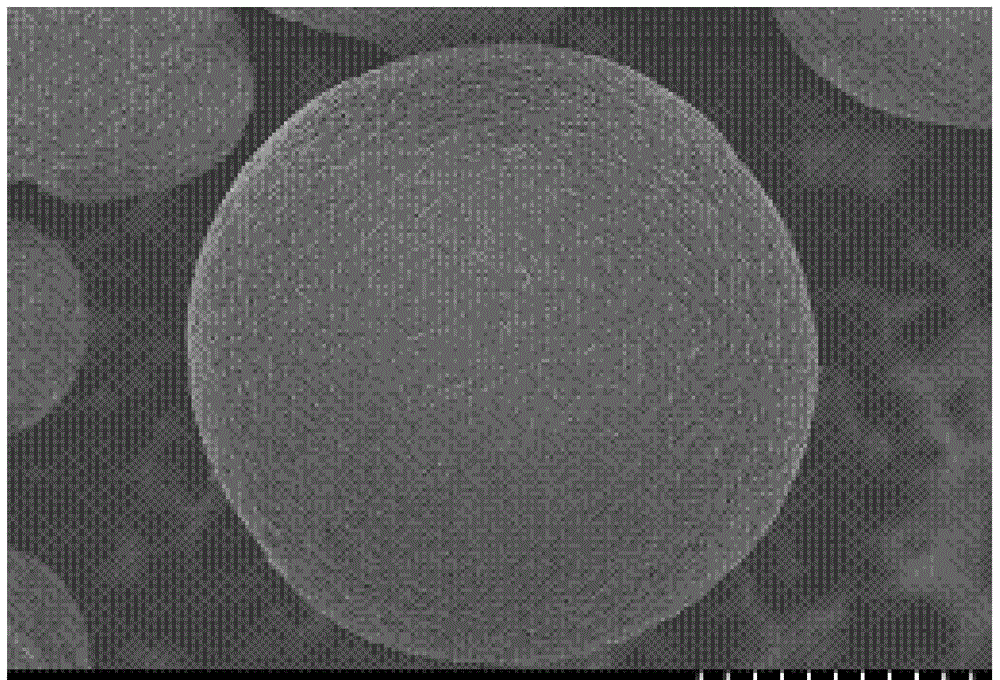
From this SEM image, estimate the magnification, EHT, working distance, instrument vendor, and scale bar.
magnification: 3.5 K X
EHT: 3 kV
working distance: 9.1 mm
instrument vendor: Hitachi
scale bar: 10000 nm